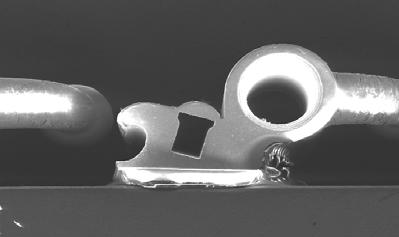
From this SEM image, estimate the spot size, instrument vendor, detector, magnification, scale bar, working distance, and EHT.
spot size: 7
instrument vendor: FEI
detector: SE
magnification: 0.035 K X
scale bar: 2000000 nm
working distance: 42.2 mm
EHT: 20 kV